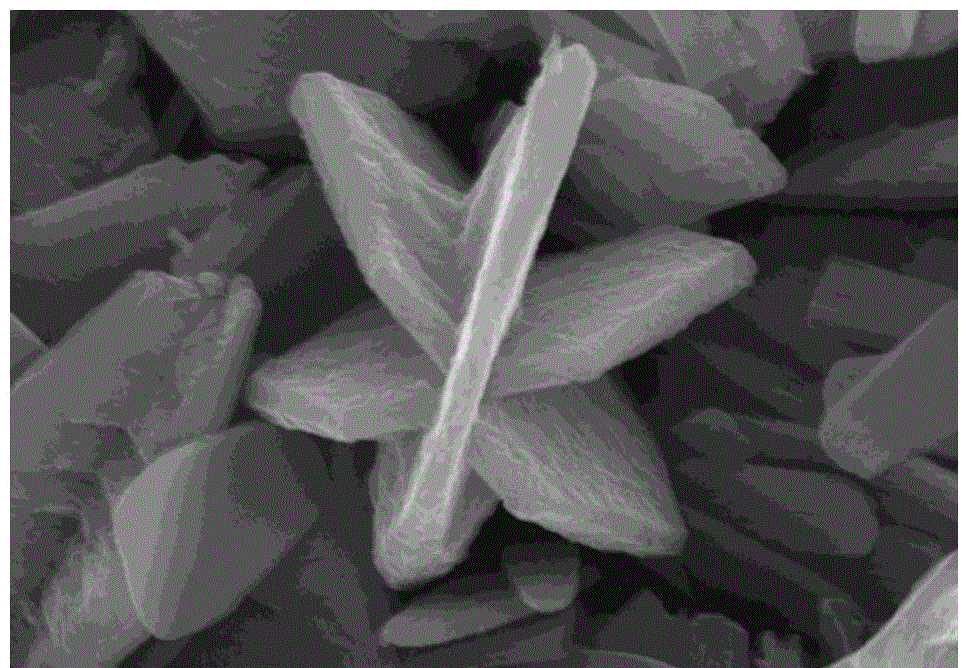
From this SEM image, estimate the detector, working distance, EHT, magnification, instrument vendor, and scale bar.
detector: SE(M)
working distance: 8.8 mm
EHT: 10 kV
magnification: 45 K X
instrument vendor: Hitachi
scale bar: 1000 nm